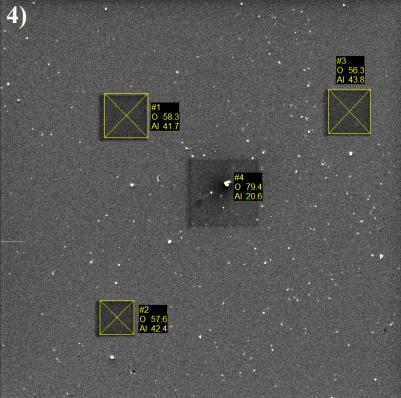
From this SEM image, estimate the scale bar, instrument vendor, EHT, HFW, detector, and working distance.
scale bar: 50000 nm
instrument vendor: Tescan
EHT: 10 kV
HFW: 220 µm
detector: SE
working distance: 15.67 mm